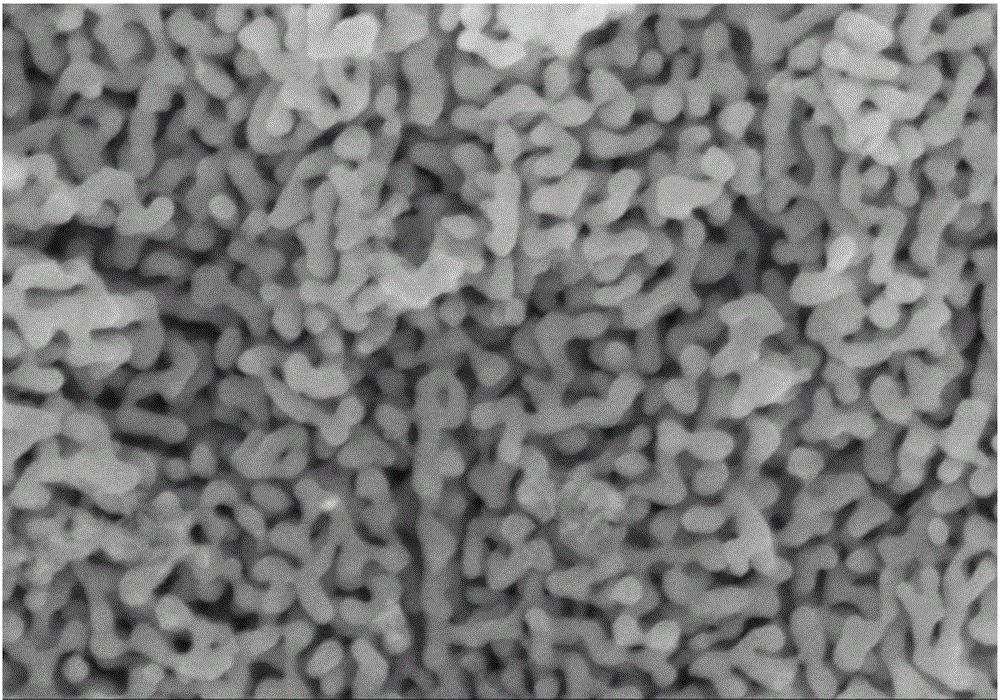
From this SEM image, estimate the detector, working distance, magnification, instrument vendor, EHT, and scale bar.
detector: SE(M)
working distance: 8.5 mm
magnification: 100 K X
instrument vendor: Hitachi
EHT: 5 kV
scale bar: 500 nm